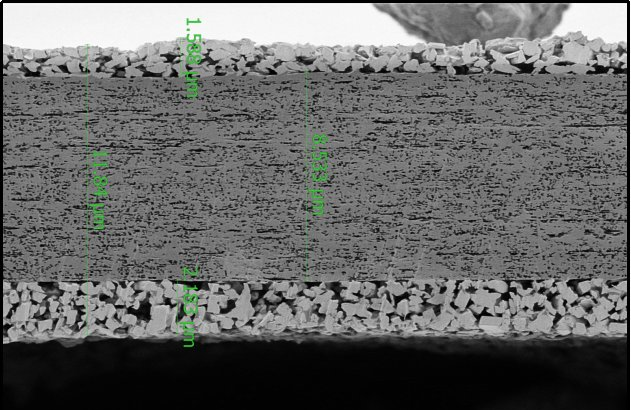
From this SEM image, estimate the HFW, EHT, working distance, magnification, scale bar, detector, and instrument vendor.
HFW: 25.4 µm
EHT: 0.5 kV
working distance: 4.5 mm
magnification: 5 K X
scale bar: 10000 nm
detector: T1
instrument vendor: Thermo